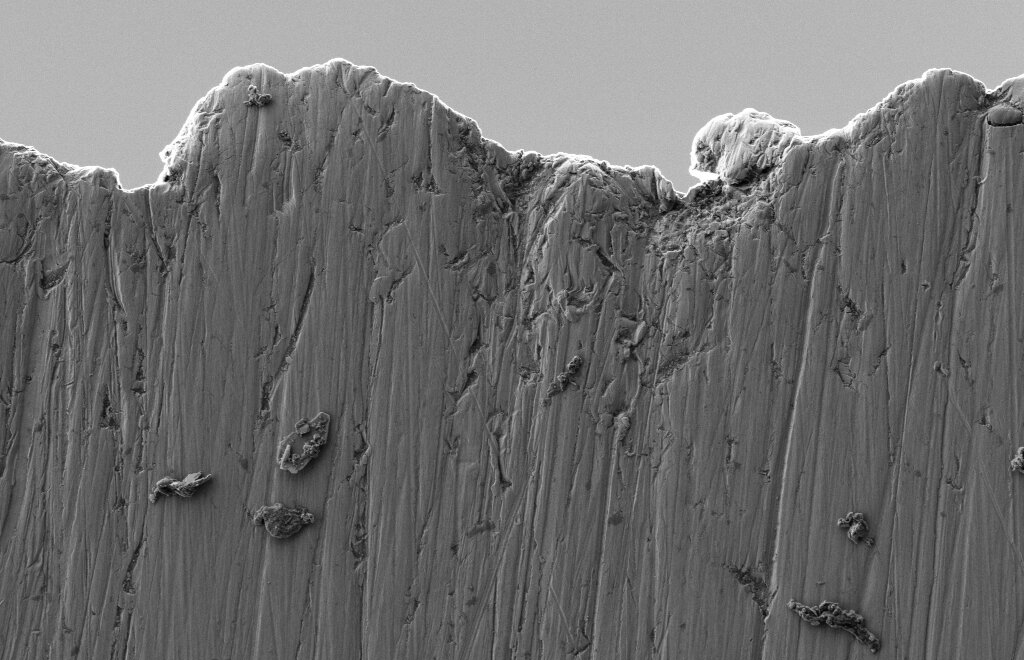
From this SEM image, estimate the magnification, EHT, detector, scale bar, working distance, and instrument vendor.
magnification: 5 K X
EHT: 1 kV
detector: SE2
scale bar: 1000 nm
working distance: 4.3 mm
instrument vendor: Zeiss